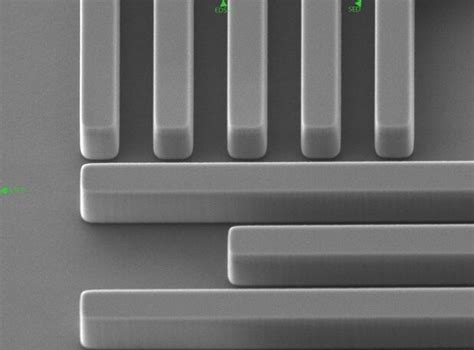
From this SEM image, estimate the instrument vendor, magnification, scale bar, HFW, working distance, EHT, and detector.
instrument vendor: JEOL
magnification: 10 K X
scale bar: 1000 nm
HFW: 12.8 µm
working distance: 8.7 mm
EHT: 3 kV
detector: SED